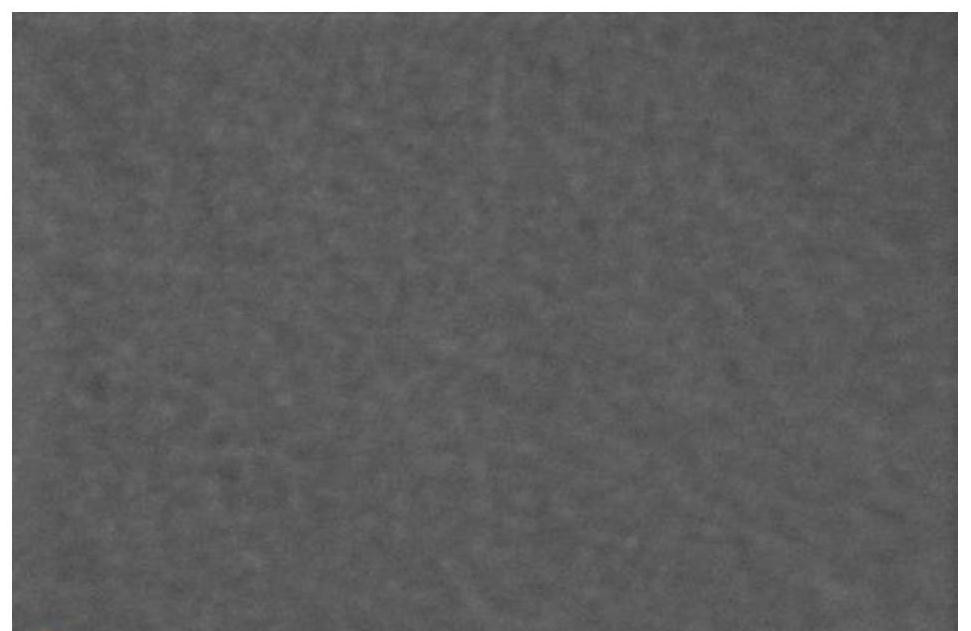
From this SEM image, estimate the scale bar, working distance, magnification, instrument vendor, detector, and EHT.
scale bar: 400 nm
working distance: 2.3 mm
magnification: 300 K X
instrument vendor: Thermo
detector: T2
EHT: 0.8984 kV